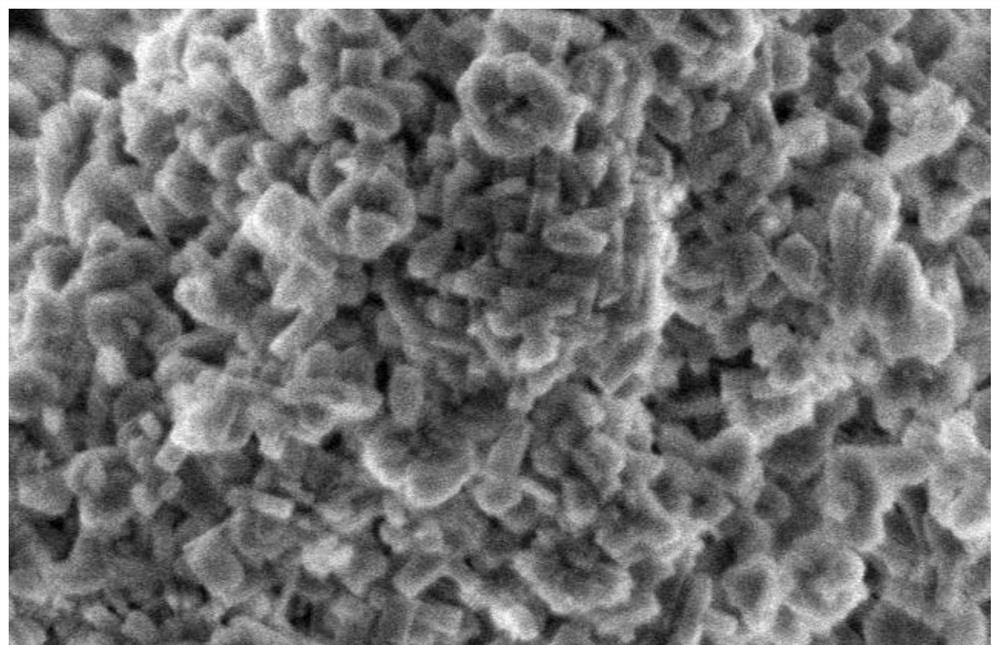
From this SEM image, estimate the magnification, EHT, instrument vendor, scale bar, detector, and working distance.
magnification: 40 K X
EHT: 2 kV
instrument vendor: Hitachi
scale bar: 1000 nm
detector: SE(M)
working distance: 9 mm